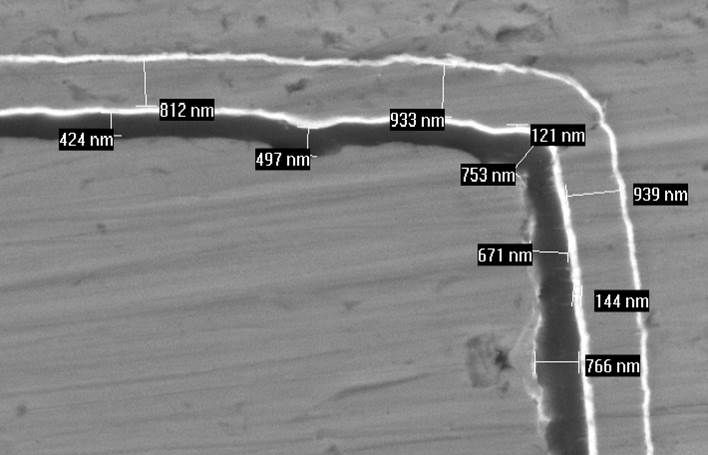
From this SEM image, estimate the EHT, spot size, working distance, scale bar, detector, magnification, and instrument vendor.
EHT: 15 kV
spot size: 3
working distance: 10 mm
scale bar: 2000 nm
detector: BSE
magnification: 10 K X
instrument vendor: FEI